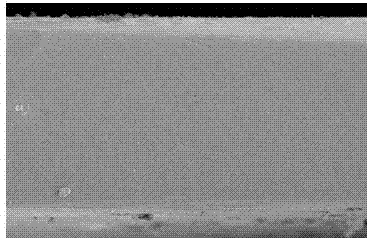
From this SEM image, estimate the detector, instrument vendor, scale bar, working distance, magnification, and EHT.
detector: SE(M)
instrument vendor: Hitachi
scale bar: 50000 nm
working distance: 9.5 mm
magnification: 0.9 K X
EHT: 3 kV